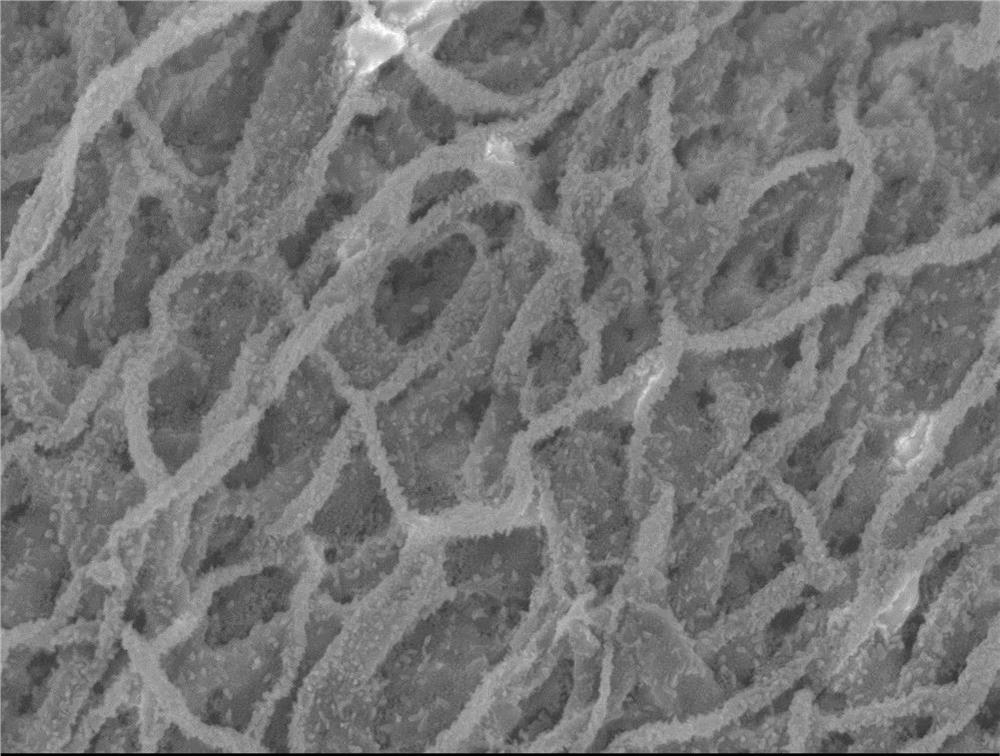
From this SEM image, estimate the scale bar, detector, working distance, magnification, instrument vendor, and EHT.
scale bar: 1000 nm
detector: SEI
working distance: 5.7 mm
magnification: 10 K X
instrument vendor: JEOL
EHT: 6 kV